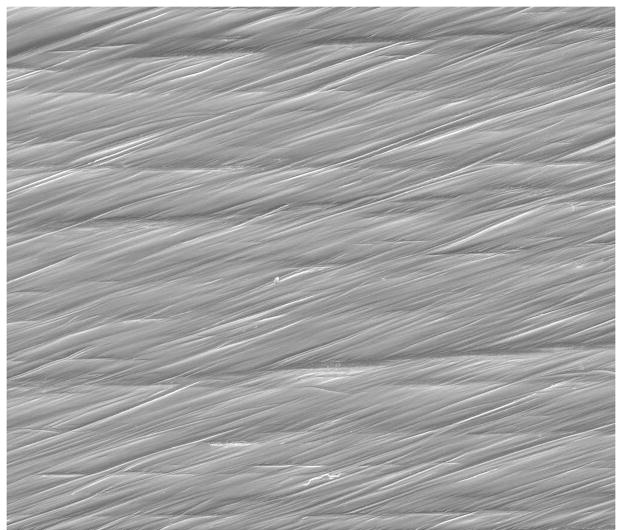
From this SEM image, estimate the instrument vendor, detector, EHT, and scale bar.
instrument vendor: FEI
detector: ETD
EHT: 20 kV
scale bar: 400000 nm